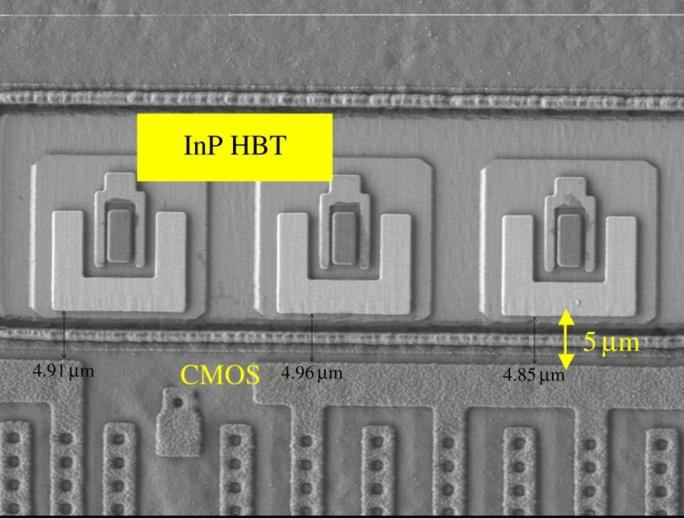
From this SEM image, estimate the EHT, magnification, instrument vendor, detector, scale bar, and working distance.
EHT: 0.7 kV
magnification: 1.7 K X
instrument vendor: JEOL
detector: LEI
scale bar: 10000 nm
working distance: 8.8 mm